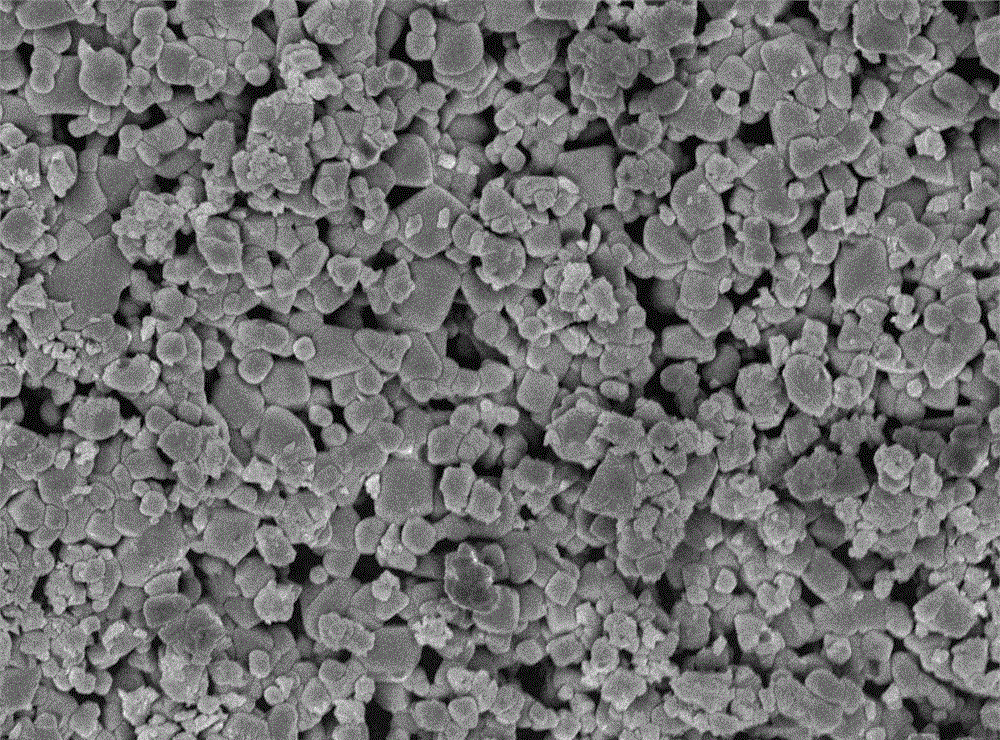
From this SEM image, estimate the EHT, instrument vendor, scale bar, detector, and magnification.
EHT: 5 kV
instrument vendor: JEOL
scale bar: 1000 nm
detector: SEI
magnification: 10 K X